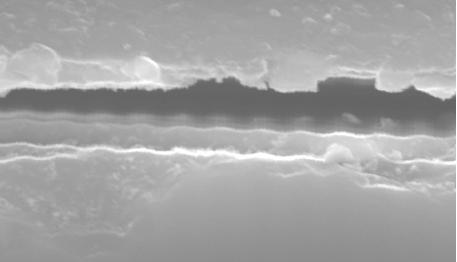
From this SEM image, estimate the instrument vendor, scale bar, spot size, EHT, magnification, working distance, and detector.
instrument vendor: FEI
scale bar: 2000 nm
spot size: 3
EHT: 20 kV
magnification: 15 K X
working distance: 11.1 mm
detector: SE